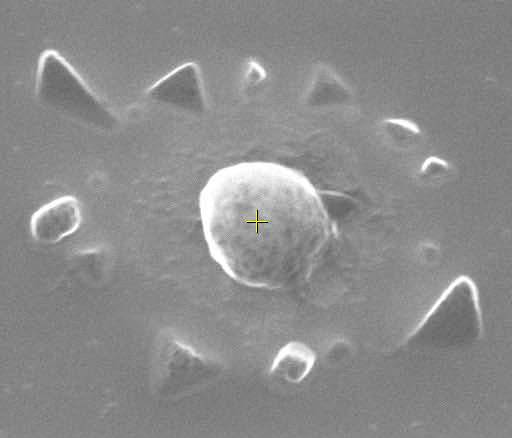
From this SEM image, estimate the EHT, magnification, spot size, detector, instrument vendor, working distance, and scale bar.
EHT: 15 kV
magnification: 60 K X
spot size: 3.5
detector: TLD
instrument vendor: FEI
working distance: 6.7 mm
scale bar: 500 nm